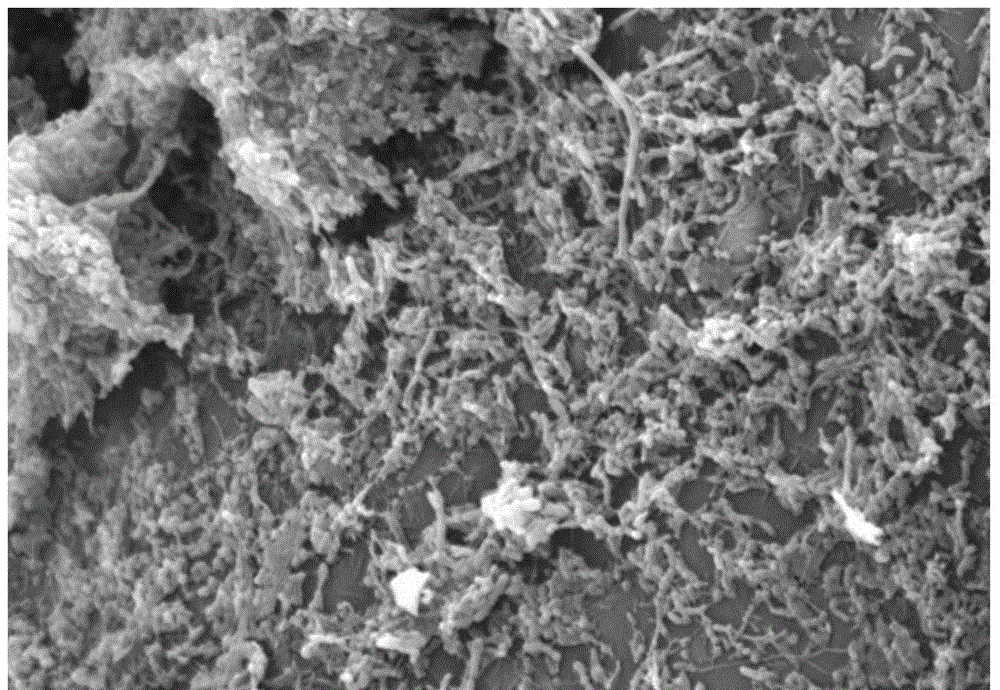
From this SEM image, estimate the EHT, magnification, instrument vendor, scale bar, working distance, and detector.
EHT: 15 kV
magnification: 2 K X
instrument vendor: Hitachi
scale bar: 20000 nm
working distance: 12.8 mm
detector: SE(M)